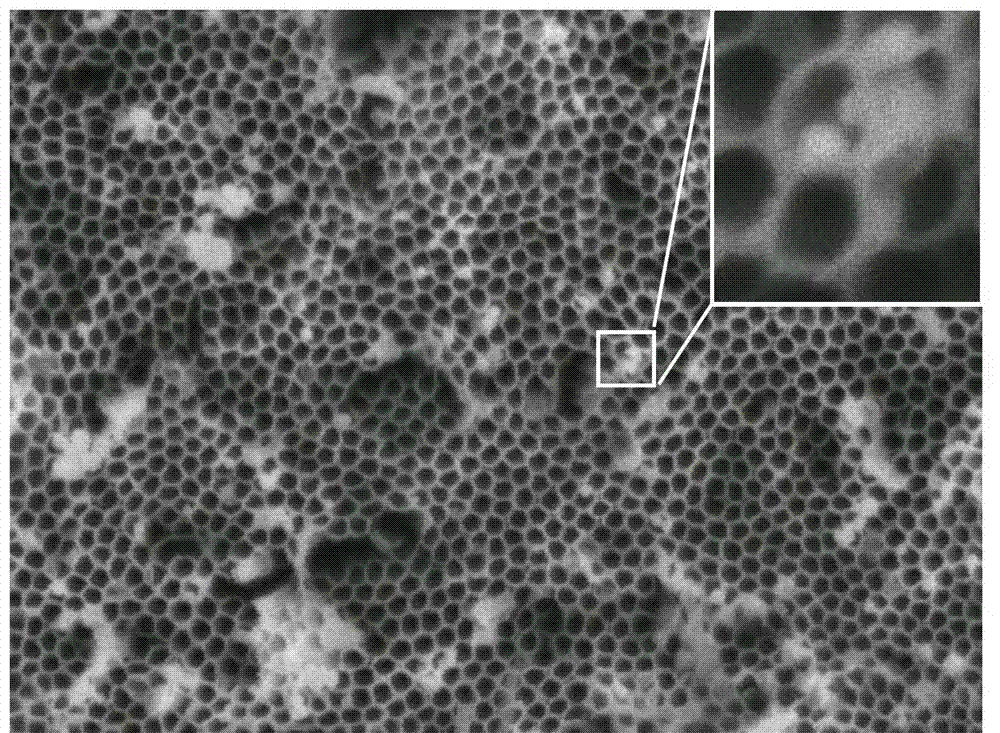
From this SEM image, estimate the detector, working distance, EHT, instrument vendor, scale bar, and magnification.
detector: SEI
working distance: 7.9 mm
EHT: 5 kV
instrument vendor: JEOL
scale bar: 100 nm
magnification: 30 K X